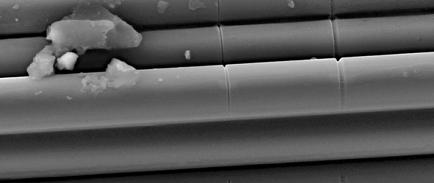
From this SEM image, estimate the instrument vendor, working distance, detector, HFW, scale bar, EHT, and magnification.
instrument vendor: Tescan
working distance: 9 mm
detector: SE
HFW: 56.2 µm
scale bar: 10000 nm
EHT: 20 kV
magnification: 4.93 K X